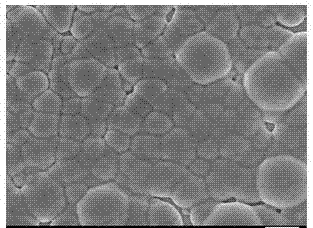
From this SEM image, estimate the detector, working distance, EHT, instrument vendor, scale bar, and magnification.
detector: SEI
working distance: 8.4 mm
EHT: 5 kV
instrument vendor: JEOL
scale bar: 1000 nm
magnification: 10 K X